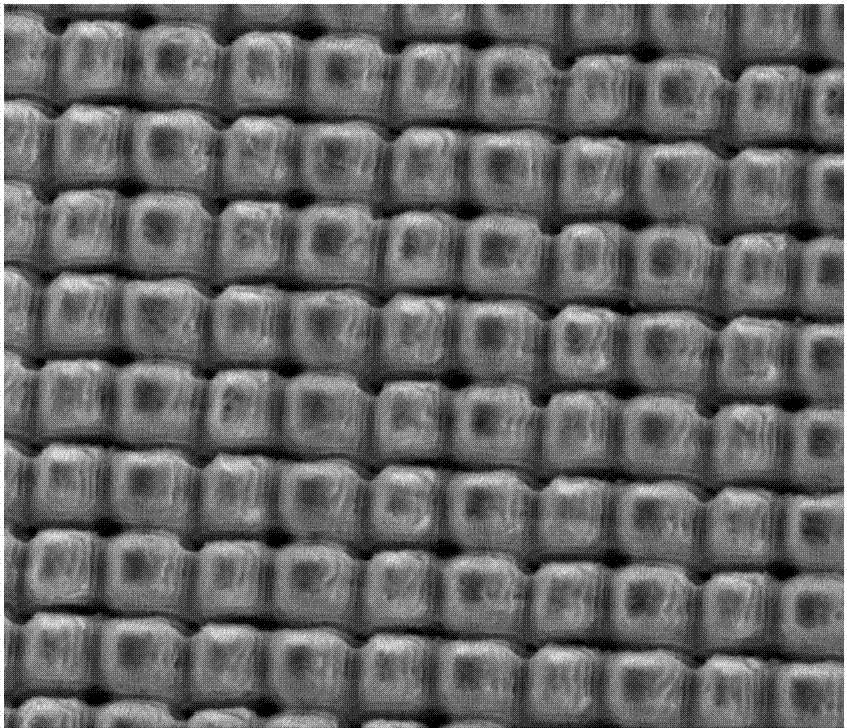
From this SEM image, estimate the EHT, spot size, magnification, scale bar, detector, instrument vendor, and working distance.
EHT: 18 kV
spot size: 4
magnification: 3 K X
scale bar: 20000 nm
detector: ETD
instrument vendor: FEI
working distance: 9 mm